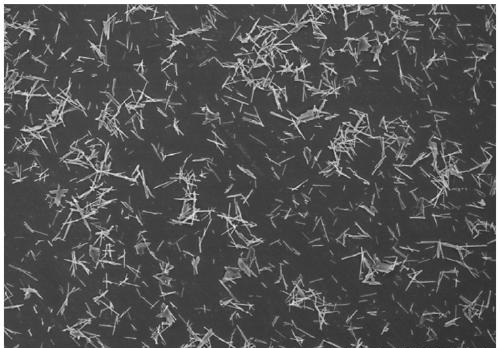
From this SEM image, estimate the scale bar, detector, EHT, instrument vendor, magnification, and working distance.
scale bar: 100000 nm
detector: SE(M)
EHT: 10 kV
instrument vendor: Hitachi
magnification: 0.4 K X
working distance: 8.8 mm